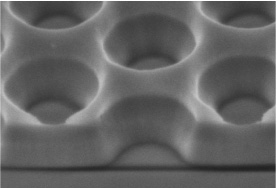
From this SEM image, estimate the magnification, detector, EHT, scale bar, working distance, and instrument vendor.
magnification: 130 K X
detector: SE(M)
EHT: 2 kV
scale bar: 400 nm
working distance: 9.7 mm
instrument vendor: Hitachi